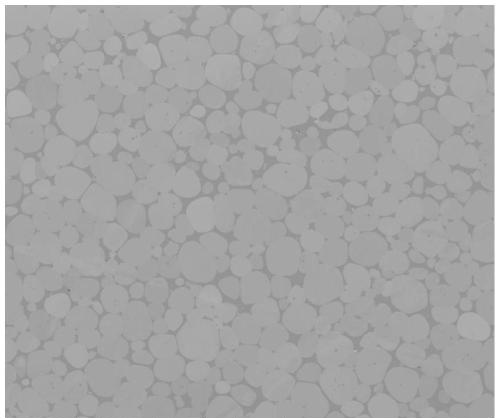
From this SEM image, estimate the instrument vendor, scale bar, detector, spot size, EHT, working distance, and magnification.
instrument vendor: FEI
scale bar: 200000 nm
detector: ETD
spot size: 6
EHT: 20 kV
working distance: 8.3 mm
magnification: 0.5 K X